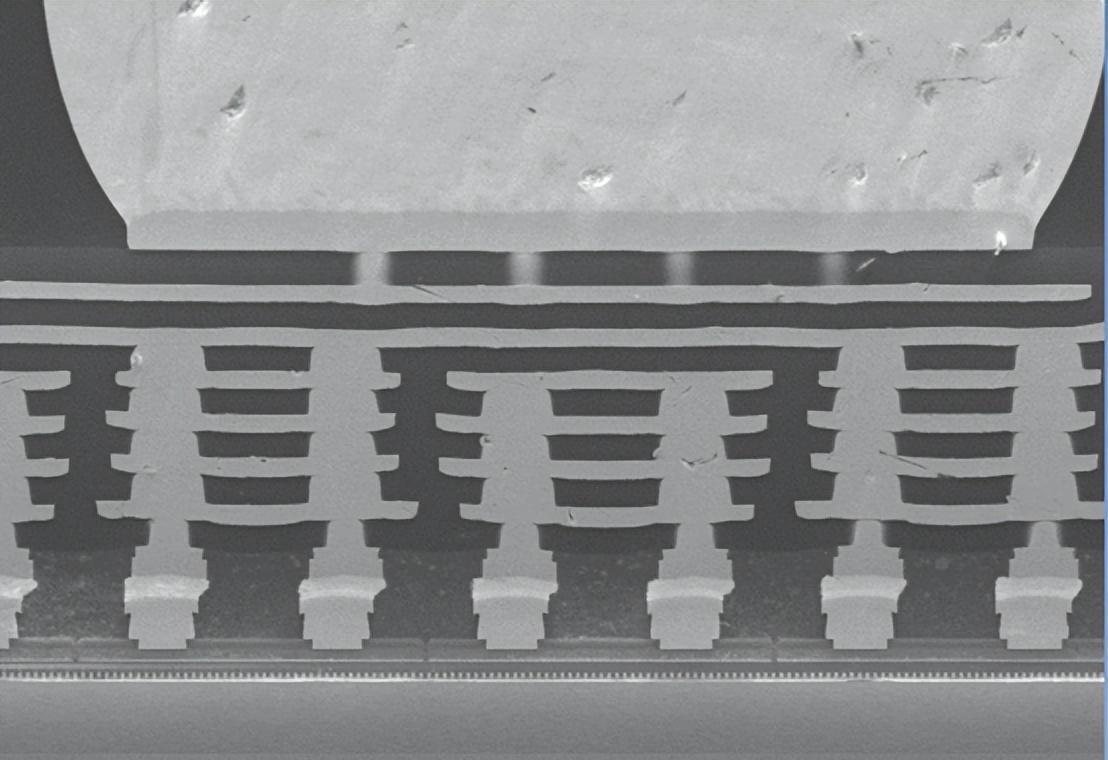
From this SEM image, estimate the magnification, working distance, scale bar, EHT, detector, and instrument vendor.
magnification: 0.5 K X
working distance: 9.8 mm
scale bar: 100000 nm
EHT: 15 kV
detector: LM(UL)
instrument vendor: Hitachi